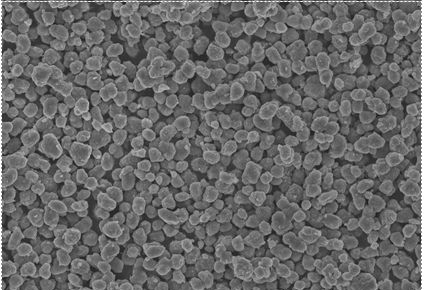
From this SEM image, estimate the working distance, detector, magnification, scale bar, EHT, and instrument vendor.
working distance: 9.6 mm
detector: SE(U)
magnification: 1 K X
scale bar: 50000 nm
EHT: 20 kV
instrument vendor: Hitachi